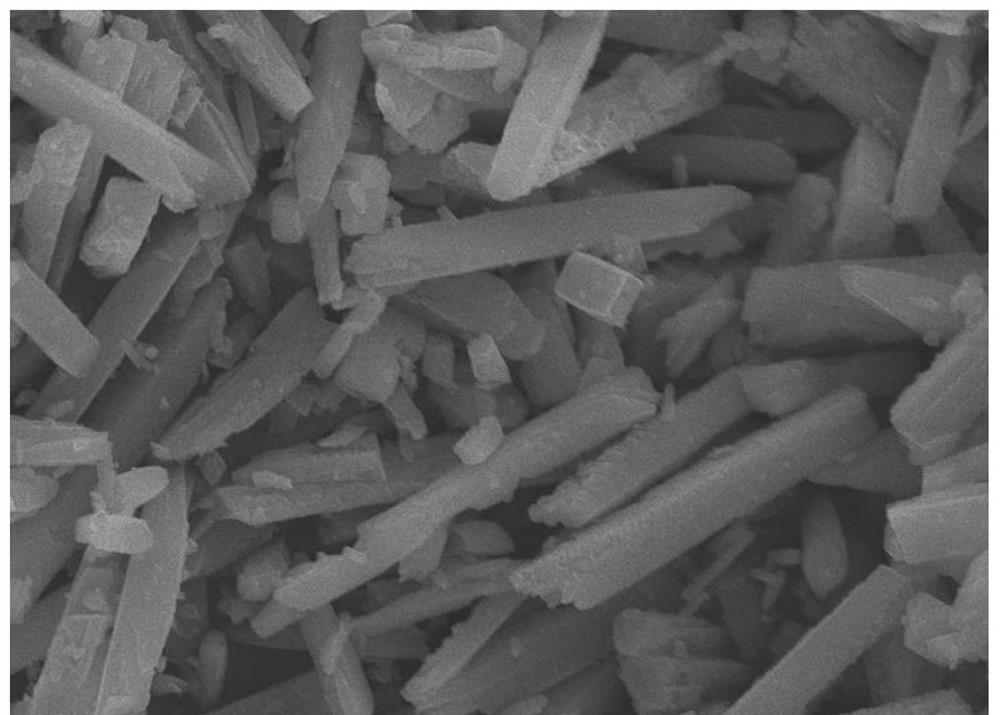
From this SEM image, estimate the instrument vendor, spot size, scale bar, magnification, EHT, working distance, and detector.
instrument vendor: JEOL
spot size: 30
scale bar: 5000 nm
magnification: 3 K X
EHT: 20 kV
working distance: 10 mm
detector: SEI